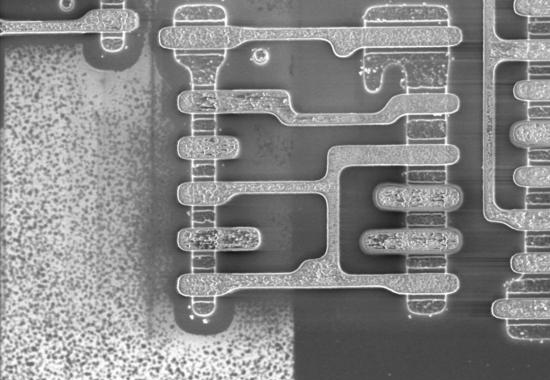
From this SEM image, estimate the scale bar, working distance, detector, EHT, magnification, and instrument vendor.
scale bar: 5000 nm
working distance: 5.1 mm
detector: SE(M)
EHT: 1.5 kV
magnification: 7 K X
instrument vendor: Hitachi